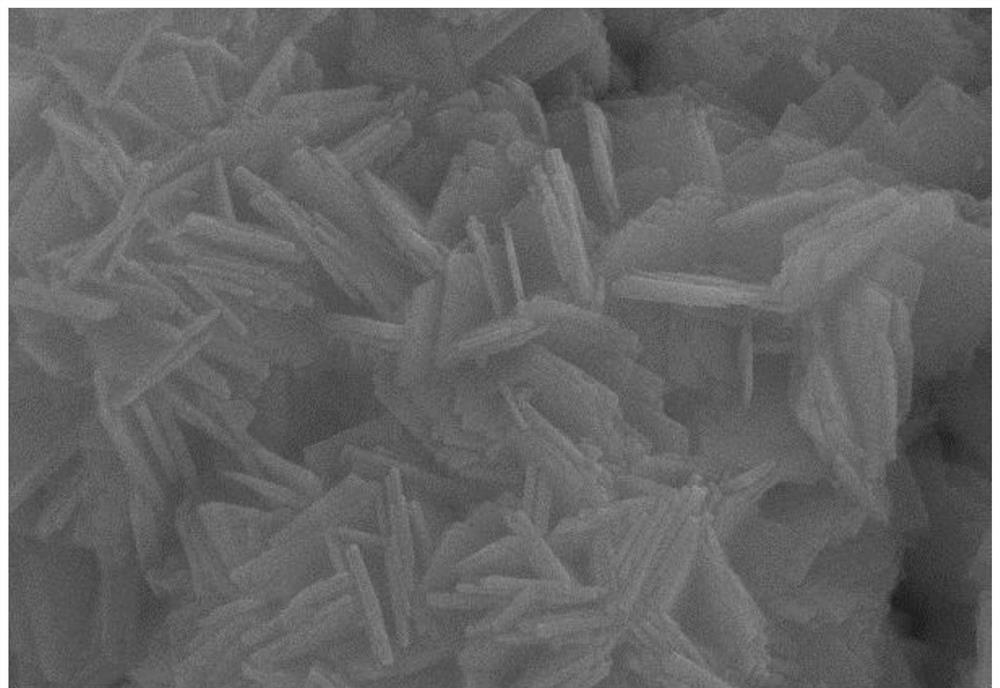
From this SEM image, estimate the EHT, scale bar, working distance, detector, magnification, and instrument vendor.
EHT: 20 kV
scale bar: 200 nm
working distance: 8 mm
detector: SE2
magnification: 20 K X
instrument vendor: Zeiss